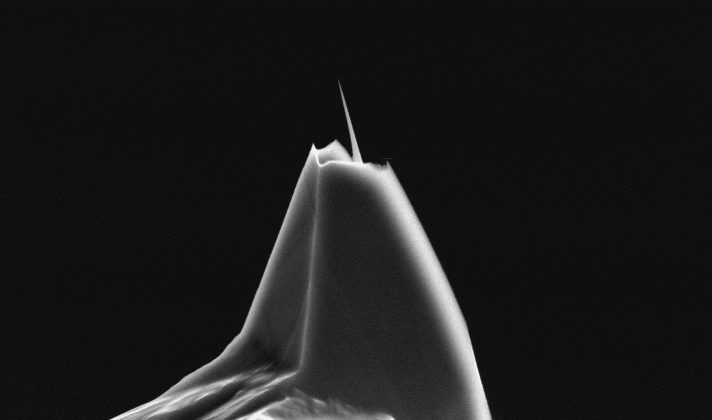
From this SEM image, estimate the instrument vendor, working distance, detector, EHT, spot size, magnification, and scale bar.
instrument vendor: FEI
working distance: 22.4 mm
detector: SE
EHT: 10 kV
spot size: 3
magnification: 5 K X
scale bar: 5000 nm